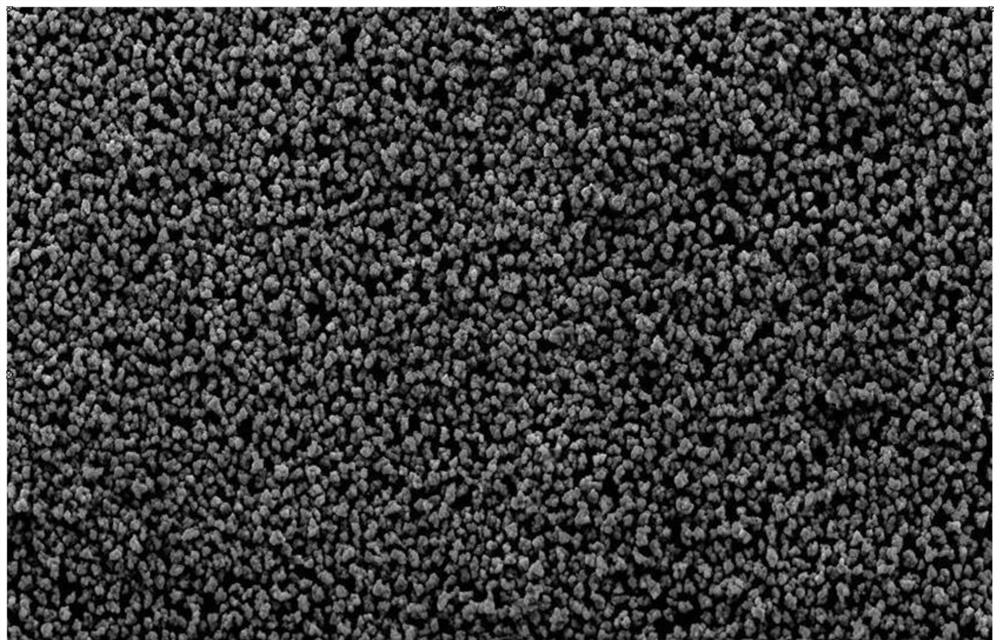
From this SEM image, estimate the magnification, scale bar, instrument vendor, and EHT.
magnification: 2 K X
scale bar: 10000 nm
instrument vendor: other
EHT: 25 kV